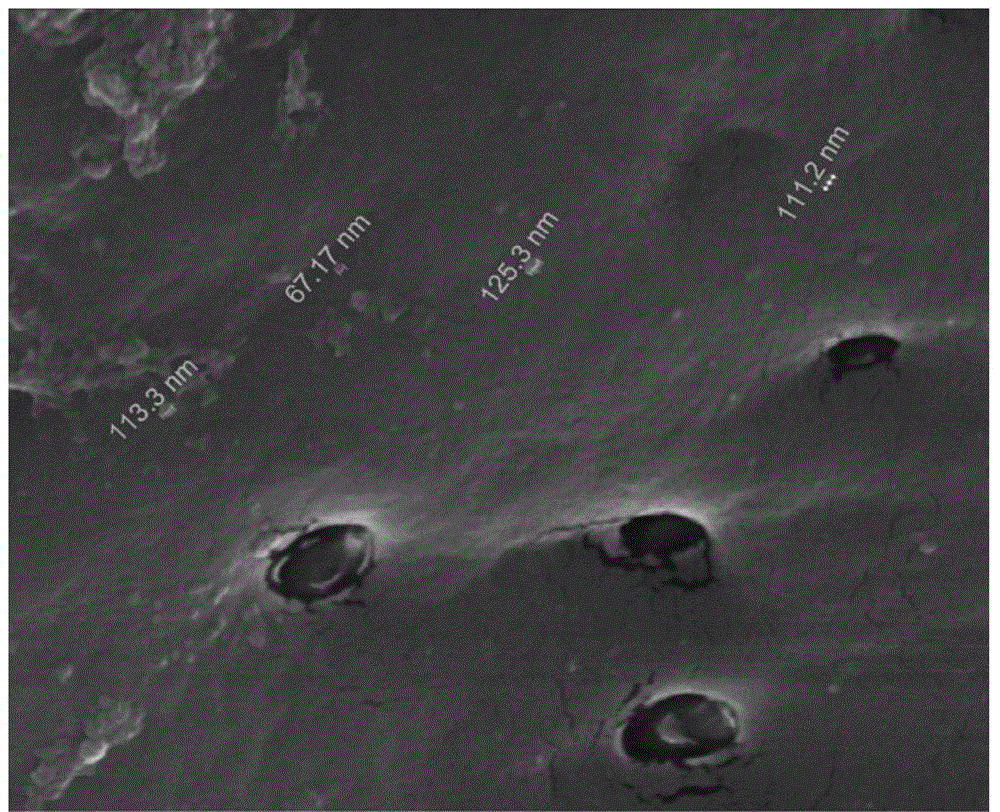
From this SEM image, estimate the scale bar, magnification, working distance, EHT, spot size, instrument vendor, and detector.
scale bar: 4000 nm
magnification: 40 K X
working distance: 4 mm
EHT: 25 kV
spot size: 3.5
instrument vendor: FEI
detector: LFD